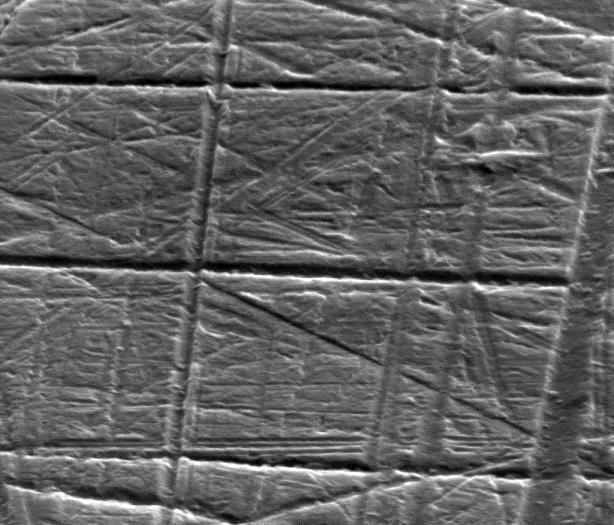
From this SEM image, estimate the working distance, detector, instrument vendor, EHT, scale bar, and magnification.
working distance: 13.2 mm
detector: ETD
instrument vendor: FEI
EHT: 30 kV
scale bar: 2000 nm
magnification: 32.5 K X